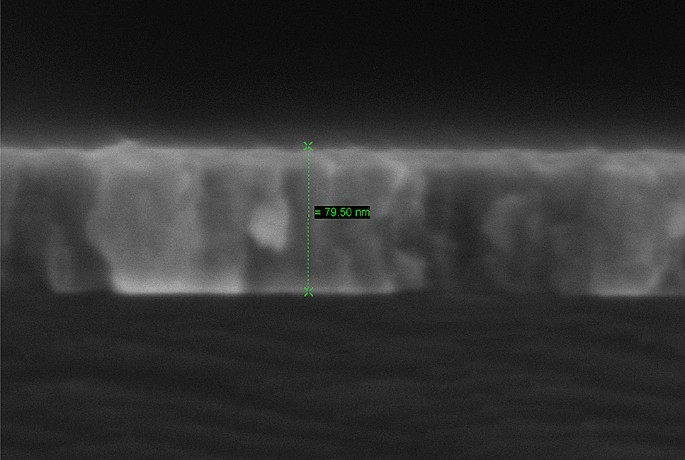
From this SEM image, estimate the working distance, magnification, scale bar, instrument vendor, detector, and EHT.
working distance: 2.2 mm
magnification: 800.79 K X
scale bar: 20 nm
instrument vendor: Zeiss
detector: InLens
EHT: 5 kV